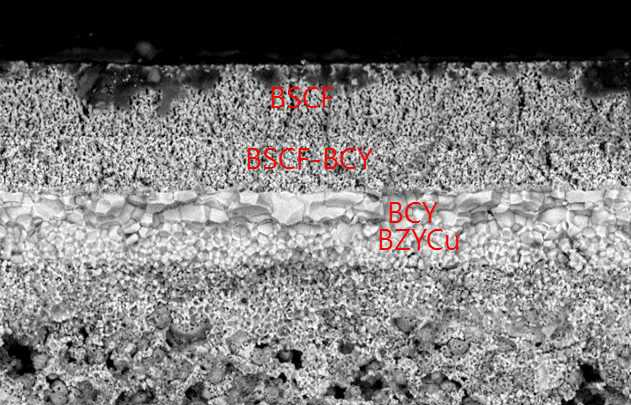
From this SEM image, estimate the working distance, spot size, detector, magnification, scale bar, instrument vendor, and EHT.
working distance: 11 mm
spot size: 3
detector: BSE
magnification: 1 K X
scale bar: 20000 nm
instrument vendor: FEI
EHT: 15 kV